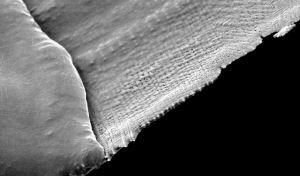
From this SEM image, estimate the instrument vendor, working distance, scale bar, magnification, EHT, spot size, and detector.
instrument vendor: FEI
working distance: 21.4 mm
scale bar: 50000 nm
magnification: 0.425 K X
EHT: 30 kV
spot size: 3.7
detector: SE